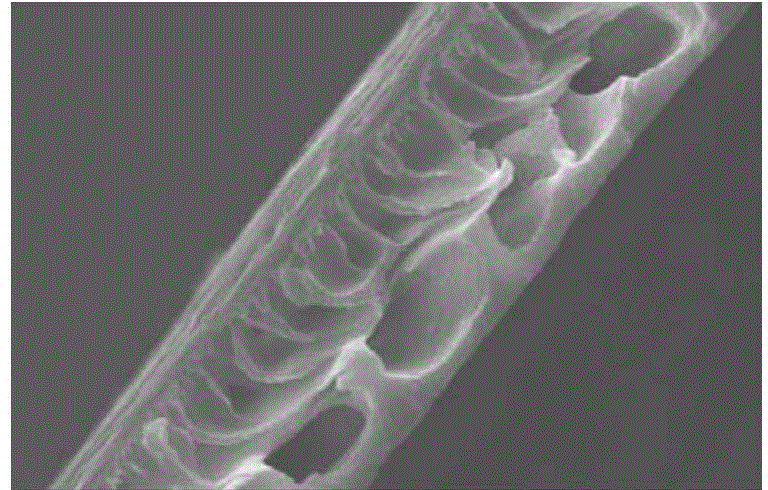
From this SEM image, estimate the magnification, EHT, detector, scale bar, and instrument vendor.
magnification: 0.3 K X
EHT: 30 kV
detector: SEI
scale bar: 50000 nm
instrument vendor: JEOL